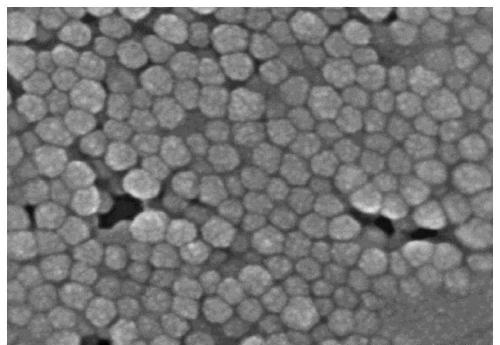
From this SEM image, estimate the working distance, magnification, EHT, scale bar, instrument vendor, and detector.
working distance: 9.9 mm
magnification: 200 K X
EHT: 5 kV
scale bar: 200 nm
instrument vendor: Hitachi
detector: SE(M)